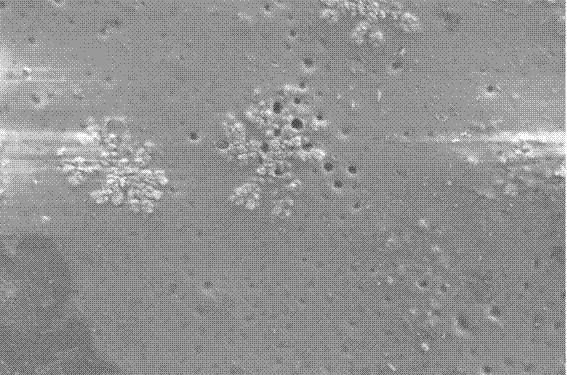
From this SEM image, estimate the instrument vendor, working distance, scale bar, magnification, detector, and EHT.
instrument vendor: Zeiss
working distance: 4.8 mm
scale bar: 2000 nm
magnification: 15 K X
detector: SE2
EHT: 1 kV